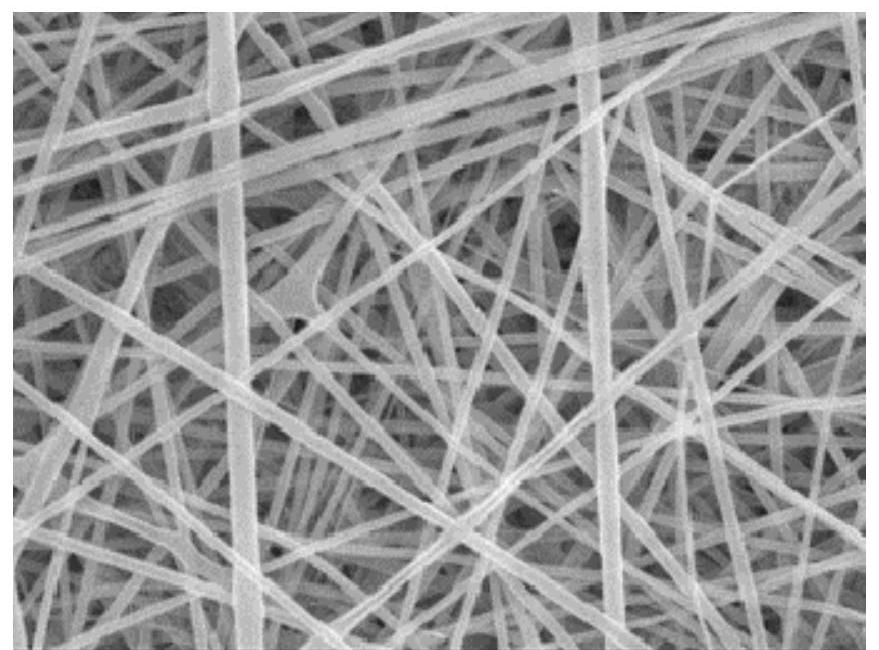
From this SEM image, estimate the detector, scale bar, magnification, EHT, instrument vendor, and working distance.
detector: SEM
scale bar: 1000 nm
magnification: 5 K X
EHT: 15 kV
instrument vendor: JEOL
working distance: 8.1 mm